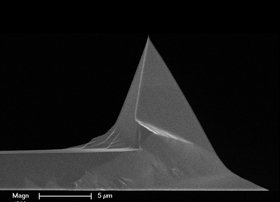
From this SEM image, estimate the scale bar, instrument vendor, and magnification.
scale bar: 5000 nm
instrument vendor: FEI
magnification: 4.5 K X